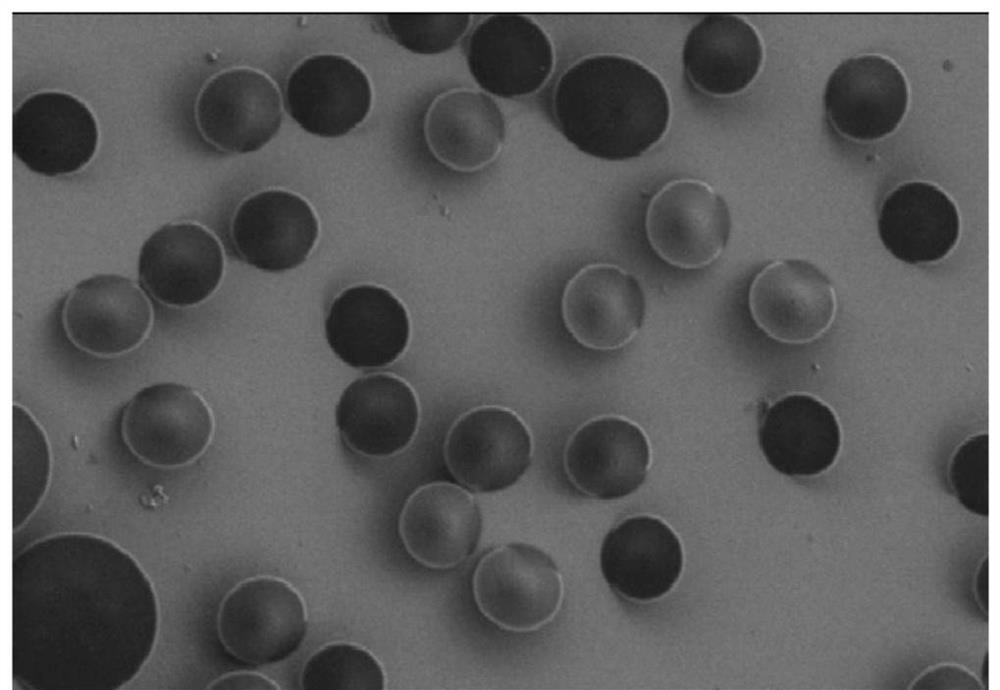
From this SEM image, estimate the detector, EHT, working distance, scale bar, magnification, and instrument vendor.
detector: SE(L)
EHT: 1 kV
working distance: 9.9 mm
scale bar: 10000 nm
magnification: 5 K X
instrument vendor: Hitachi